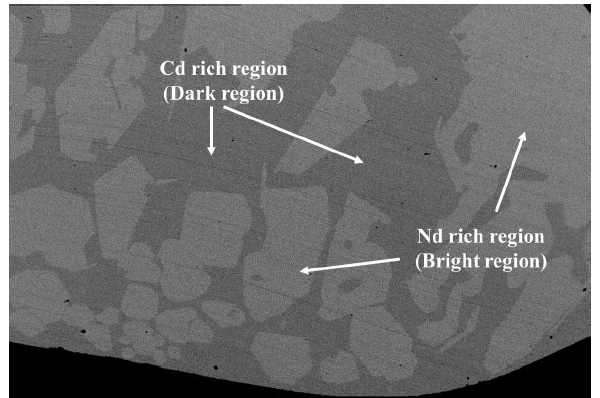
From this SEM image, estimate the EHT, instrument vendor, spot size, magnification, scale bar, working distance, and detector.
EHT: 20 kV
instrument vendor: JEOL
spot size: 14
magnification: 0.06 K X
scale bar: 100000 nm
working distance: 11.2 mm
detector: BSEI